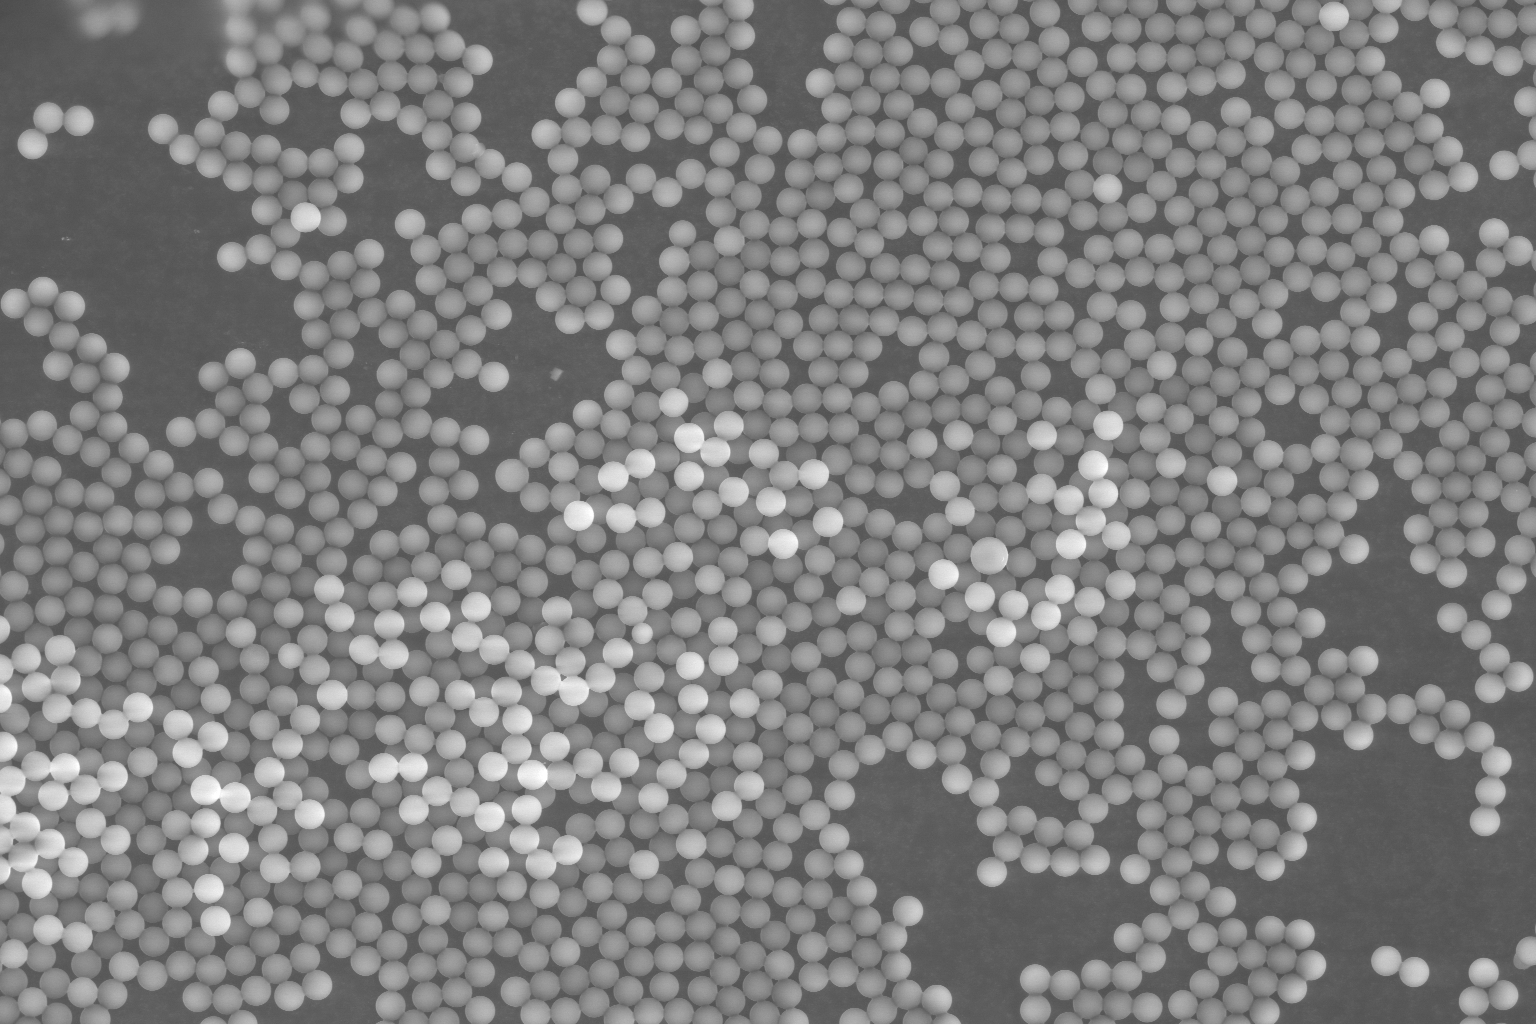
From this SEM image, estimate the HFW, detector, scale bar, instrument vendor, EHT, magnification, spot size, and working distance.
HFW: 414 µm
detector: SE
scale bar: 100000 nm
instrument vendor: FEI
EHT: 20 kV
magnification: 1 K X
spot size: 5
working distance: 11.3 mm